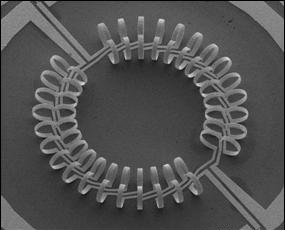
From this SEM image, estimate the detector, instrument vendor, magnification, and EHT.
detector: SE(M)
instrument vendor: Hitachi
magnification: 0.04 K X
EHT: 20 kV